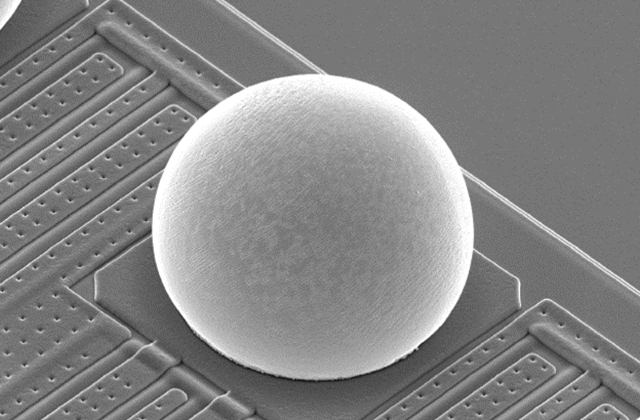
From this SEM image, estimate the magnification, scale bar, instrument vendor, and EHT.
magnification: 1 K X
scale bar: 10000 nm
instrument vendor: JEOL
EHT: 10 kV